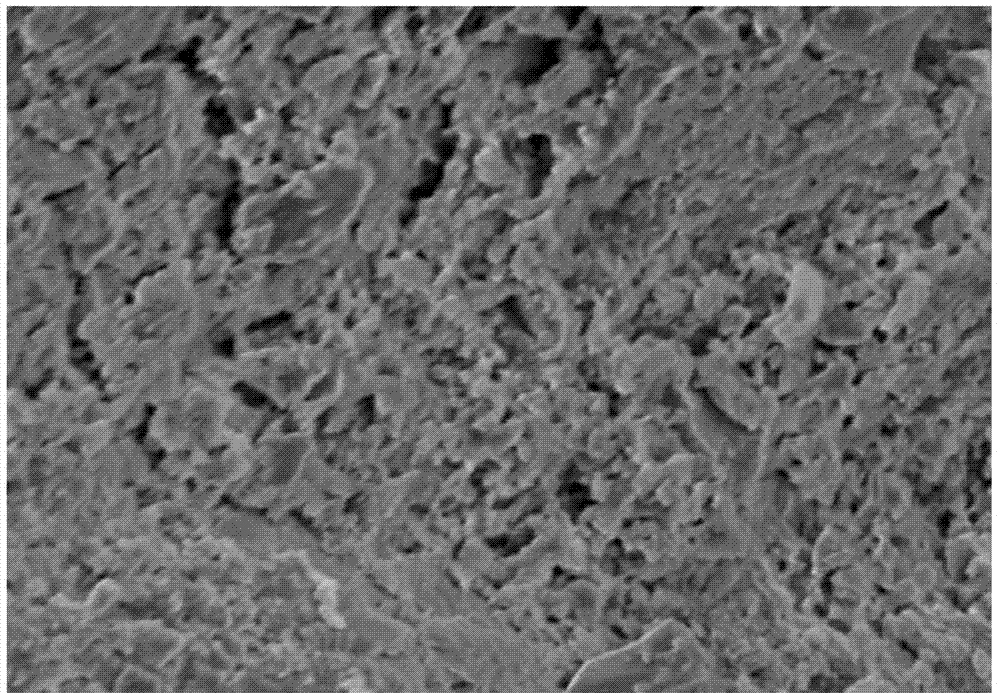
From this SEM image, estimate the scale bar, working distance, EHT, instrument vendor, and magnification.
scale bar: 10000 nm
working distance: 15.7 mm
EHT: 5 kV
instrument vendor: Hitachi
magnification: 5 K X